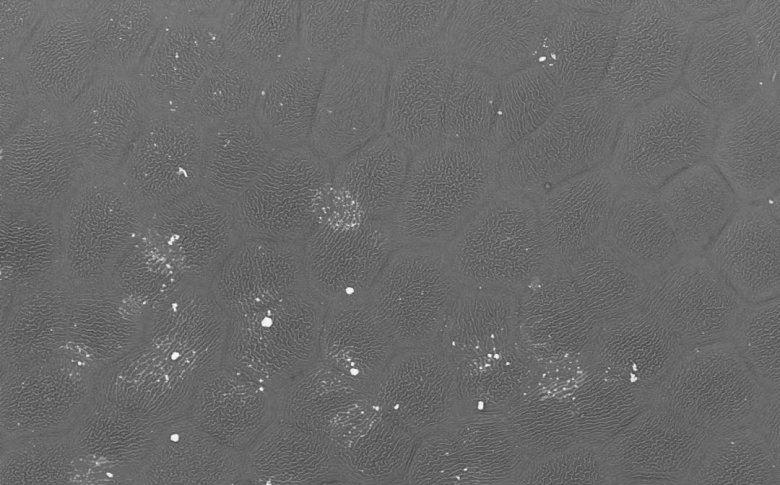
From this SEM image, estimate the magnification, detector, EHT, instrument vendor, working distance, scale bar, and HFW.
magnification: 0.225 K X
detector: BSD Full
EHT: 10 kV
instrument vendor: Thermo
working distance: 7.497 mm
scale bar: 150000 nm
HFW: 563 µm